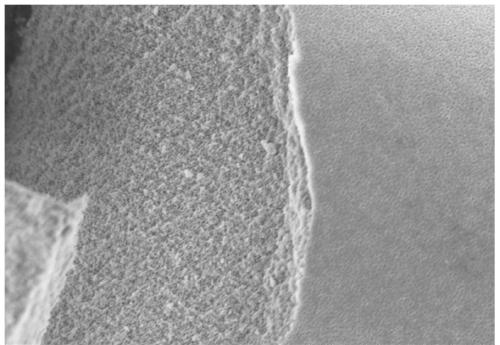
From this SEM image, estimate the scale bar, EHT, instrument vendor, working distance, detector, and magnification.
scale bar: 5000 nm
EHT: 5 kV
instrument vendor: Hitachi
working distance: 9.6 mm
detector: SE(M)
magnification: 9 K X